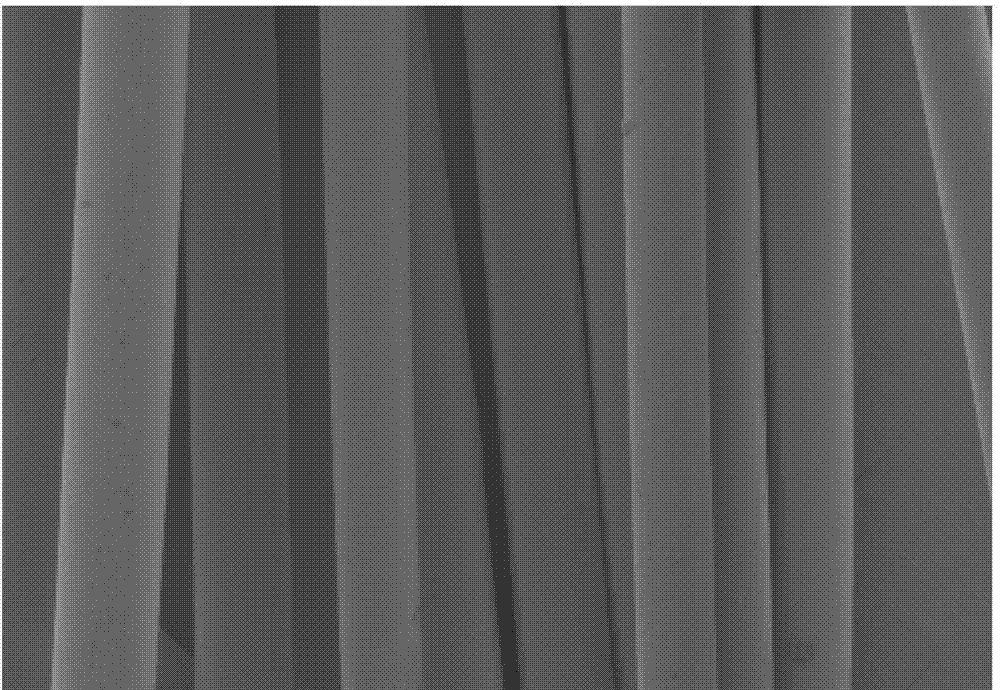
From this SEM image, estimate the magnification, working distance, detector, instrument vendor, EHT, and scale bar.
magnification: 0.2 K X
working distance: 9.1 mm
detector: LM(L)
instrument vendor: Hitachi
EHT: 1 kV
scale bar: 200000 nm